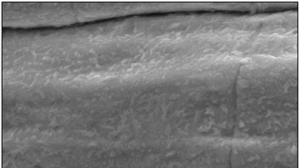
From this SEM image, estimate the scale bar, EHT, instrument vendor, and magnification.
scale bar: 5000 nm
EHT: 20 kV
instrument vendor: JEOL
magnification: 5 K X